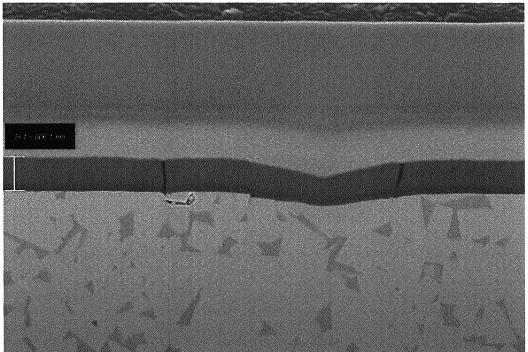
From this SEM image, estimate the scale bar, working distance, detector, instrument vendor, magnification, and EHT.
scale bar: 1000 nm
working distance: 5.1 mm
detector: InLens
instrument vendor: Zeiss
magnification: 8.14 K X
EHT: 5 kV